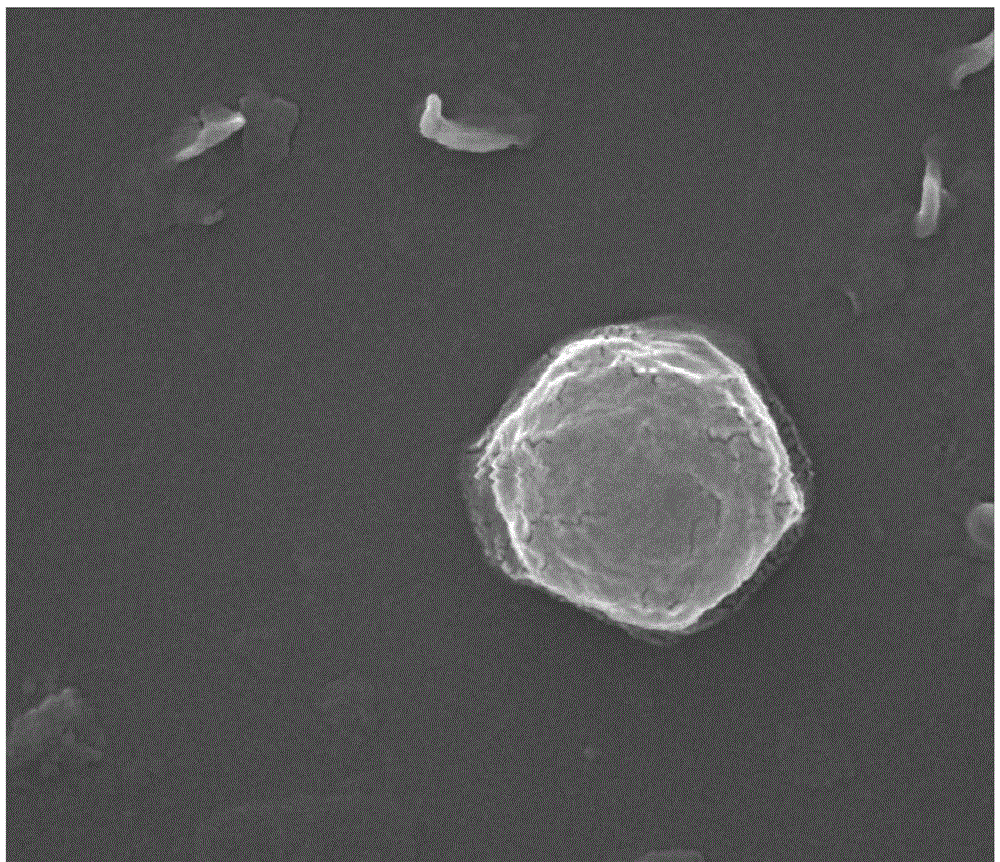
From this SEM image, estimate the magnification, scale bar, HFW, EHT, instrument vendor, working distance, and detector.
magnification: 80 K X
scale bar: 1000 nm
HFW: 3.37 µm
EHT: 20 kV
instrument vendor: FEI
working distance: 10 mm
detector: ETD